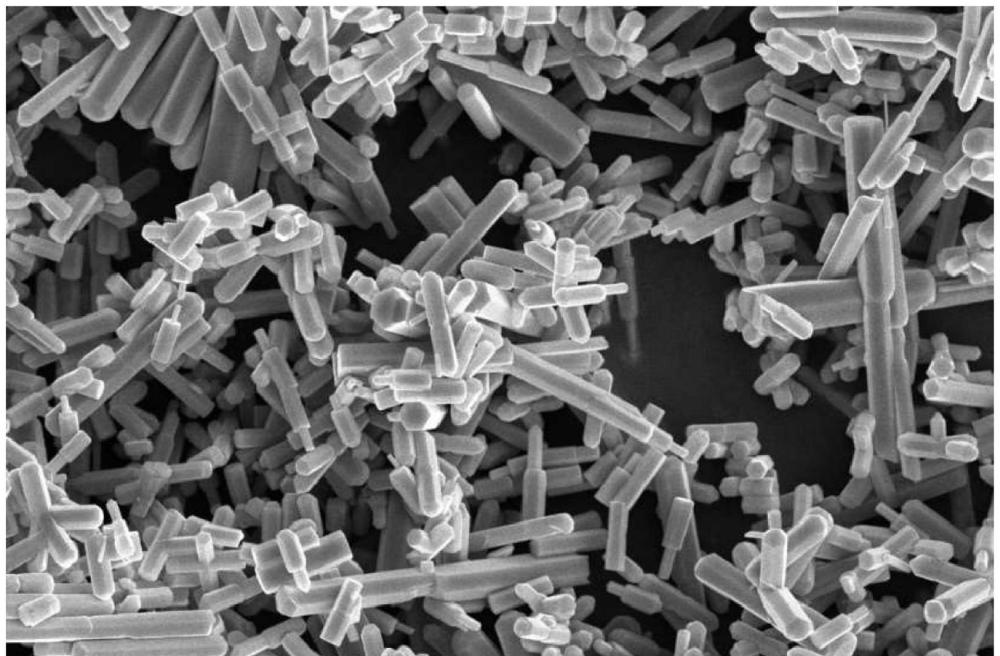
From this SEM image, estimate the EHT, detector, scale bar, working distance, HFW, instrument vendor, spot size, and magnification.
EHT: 10 kV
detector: T2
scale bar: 5000 nm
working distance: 9.9 mm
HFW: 25.4 µm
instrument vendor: Thermo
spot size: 7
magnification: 5 K X